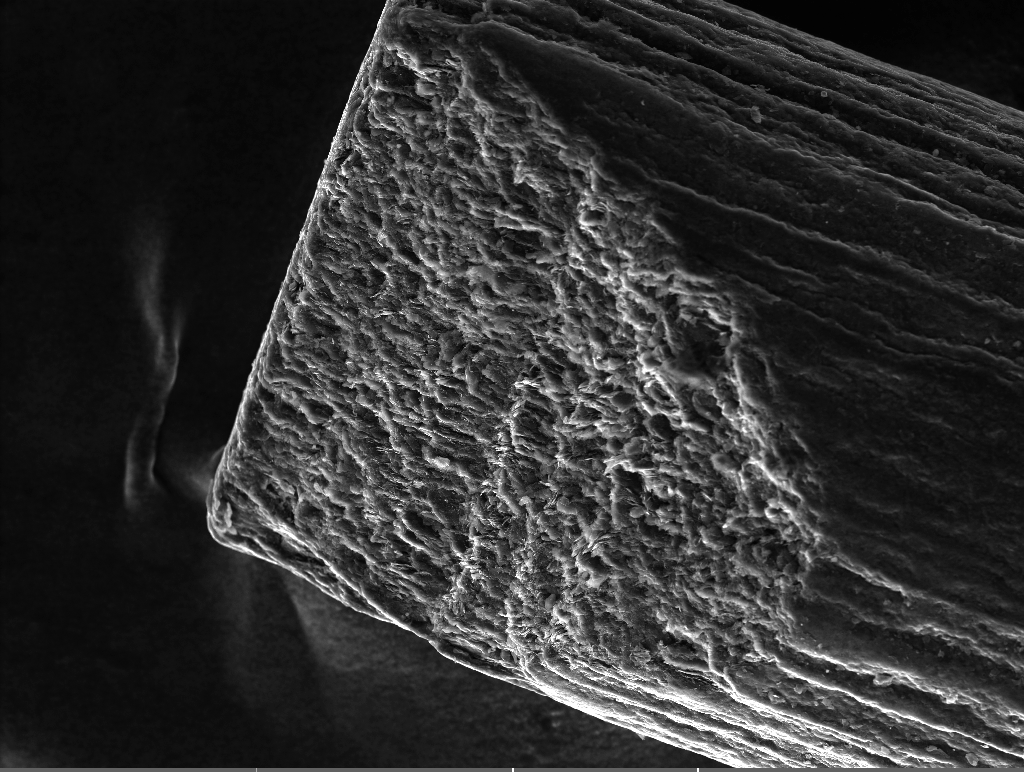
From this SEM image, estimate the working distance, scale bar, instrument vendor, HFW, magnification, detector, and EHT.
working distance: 15 mm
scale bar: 100000 nm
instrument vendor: Tescan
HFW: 554 µm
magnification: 0.5 K X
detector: SE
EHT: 15 kV